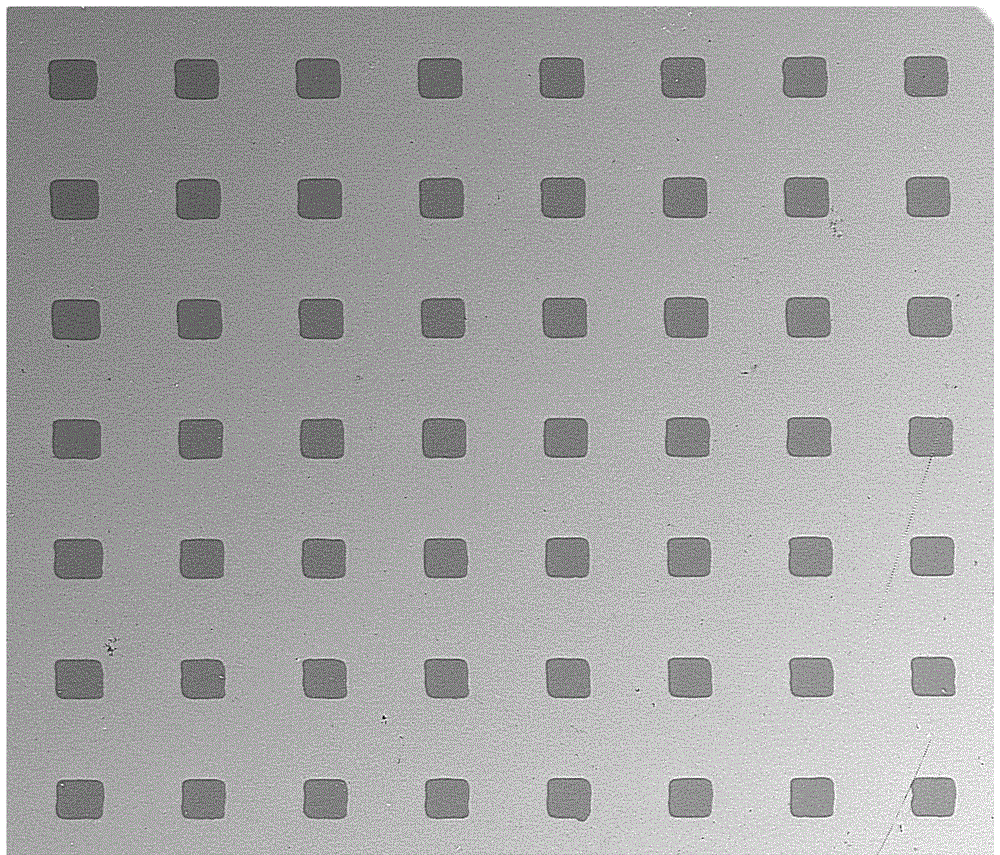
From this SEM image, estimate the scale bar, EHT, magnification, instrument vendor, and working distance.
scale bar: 500000 nm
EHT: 10 kV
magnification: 0.106 K X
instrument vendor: FEI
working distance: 4.2 mm